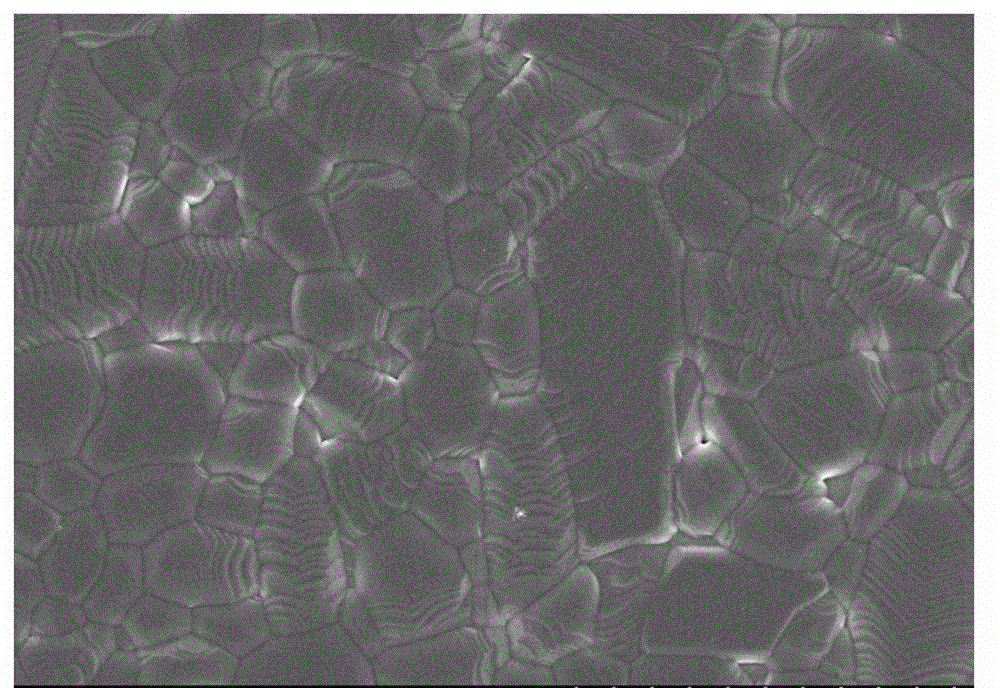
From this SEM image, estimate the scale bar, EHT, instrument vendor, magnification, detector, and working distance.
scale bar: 10000 nm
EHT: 5 kV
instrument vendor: Hitachi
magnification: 5 K X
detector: SE(U,LA0)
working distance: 12.2 mm